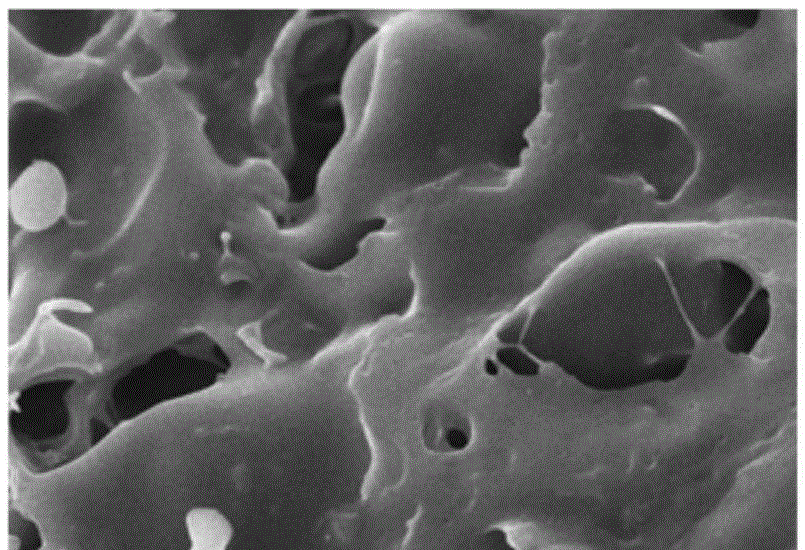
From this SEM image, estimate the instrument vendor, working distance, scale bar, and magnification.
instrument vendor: Hitachi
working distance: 10.8 mm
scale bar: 10000 nm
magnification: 3 K X